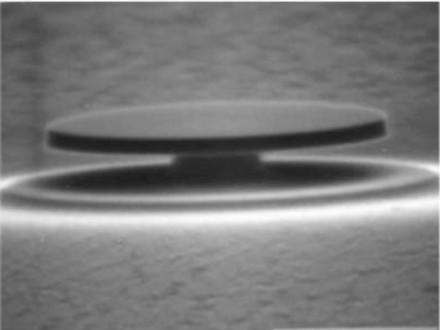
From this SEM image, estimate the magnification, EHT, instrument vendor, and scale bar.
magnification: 20 K X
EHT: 25 kV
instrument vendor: JEOL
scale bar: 2000 nm